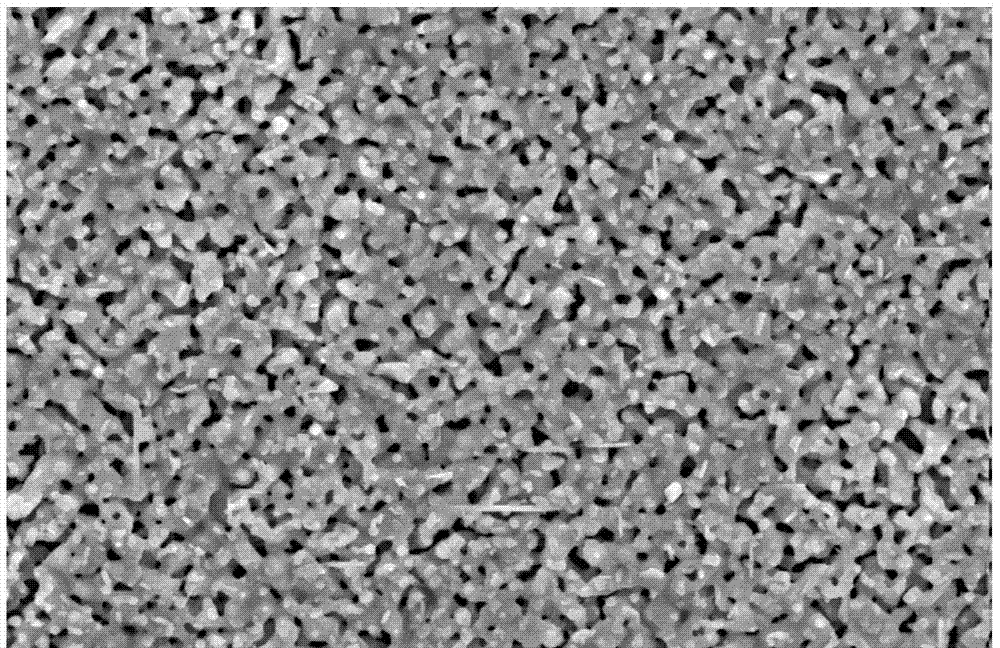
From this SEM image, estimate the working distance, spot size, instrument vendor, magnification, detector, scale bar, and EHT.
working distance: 4.6 mm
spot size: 4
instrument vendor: FEI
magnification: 20 K X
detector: TLD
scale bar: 2000 nm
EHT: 10 kV